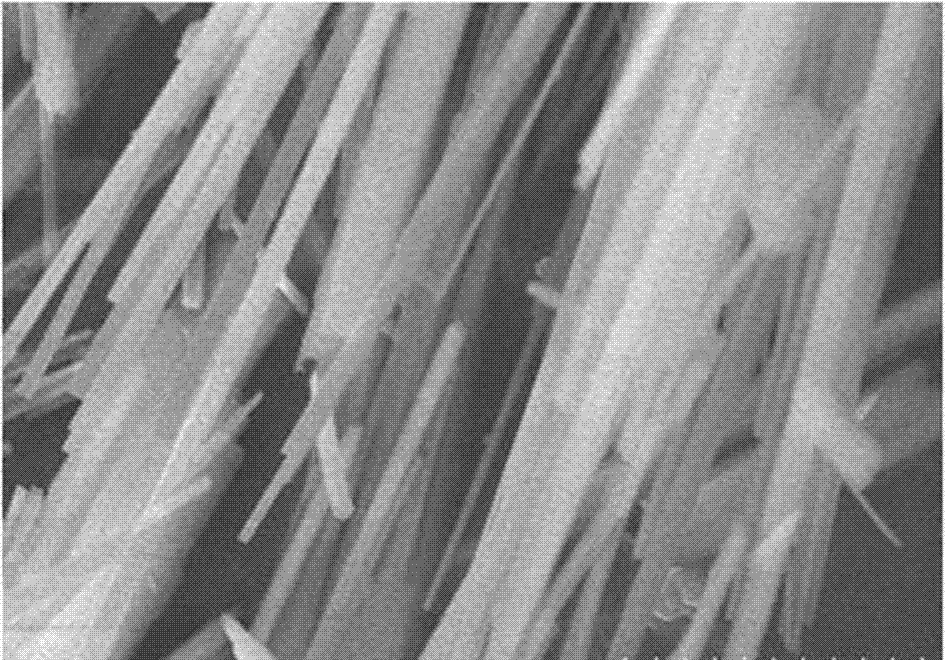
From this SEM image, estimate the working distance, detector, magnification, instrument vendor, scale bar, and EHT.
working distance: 9 mm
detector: SE(M)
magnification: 20 K X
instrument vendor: Hitachi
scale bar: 2000 nm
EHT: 10 kV